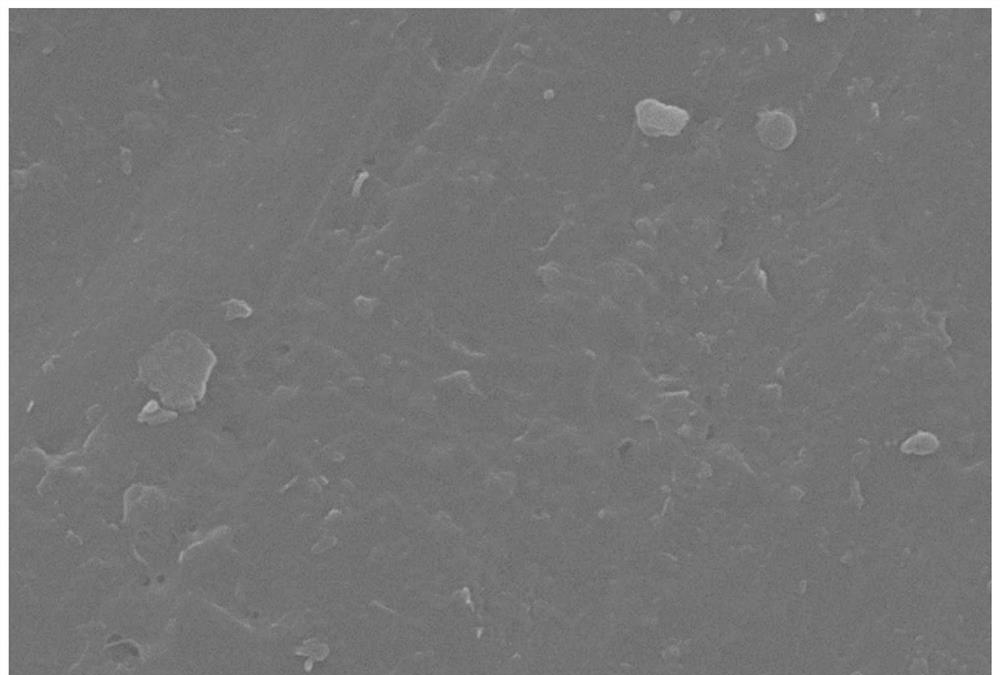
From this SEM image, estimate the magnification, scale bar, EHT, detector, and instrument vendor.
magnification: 10 K X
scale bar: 1000 nm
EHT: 30 kV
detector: SEI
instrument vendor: JEOL